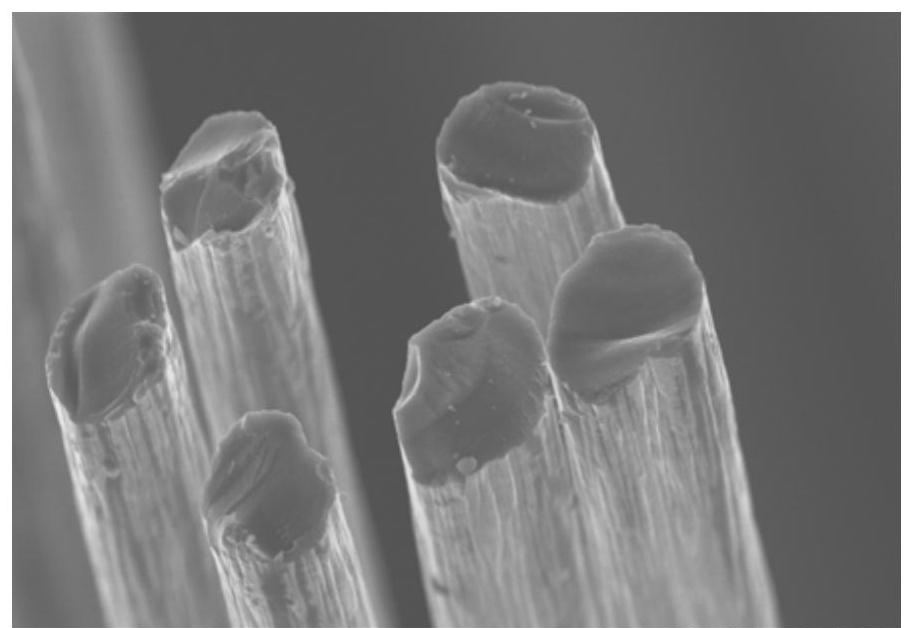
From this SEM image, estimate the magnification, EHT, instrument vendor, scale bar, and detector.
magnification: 3 K X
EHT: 8 kV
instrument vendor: Hitachi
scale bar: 10000 nm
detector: SE(M)